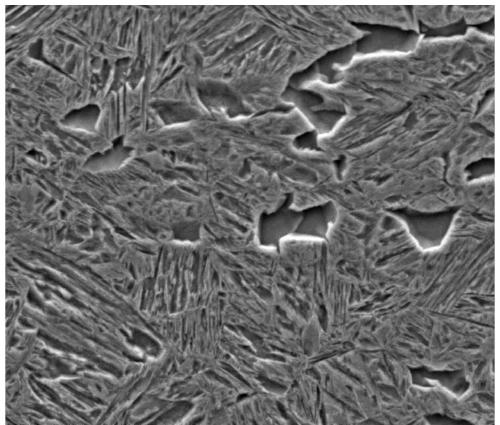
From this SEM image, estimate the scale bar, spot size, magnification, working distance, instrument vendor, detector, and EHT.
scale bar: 20000 nm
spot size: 5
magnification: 5 K X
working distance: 10 mm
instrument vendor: FEI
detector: ETD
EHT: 20 kV